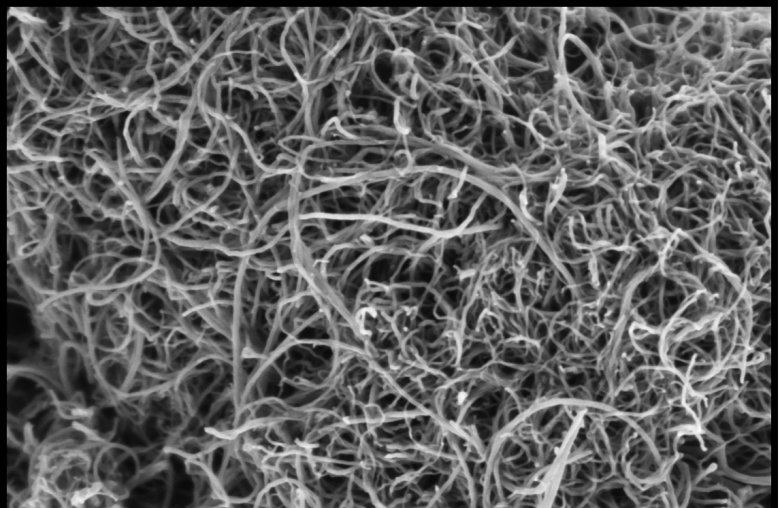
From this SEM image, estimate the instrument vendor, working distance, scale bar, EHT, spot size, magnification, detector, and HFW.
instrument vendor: Thermo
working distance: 4 mm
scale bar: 500 nm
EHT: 1 kV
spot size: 6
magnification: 50 K X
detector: T2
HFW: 2.54 µm